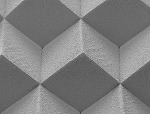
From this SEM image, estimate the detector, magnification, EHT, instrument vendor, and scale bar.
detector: Sec.High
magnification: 0.05 K X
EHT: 10 kV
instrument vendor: JEOL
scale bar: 500000 nm